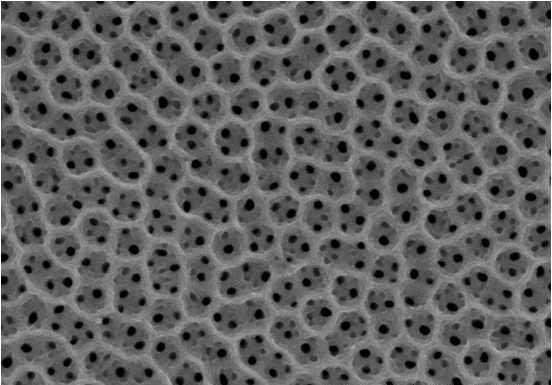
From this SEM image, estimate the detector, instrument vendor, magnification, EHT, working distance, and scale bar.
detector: SE(M)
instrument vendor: Hitachi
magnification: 50 K X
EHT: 15 kV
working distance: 19.7 mm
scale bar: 1000 nm